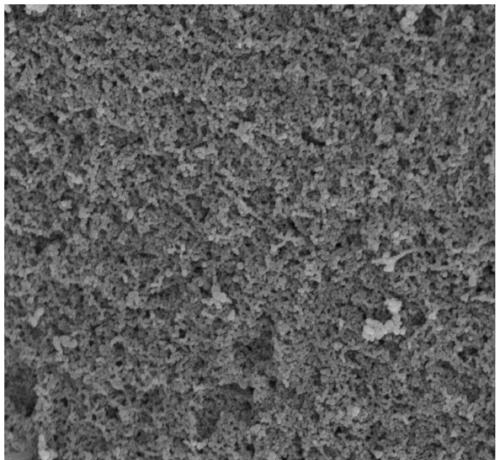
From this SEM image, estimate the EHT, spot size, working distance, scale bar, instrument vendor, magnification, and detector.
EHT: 12.5 kV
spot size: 4.5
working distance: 9.5 mm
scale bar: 5000 nm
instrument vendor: FEI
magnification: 5 K X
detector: ETD